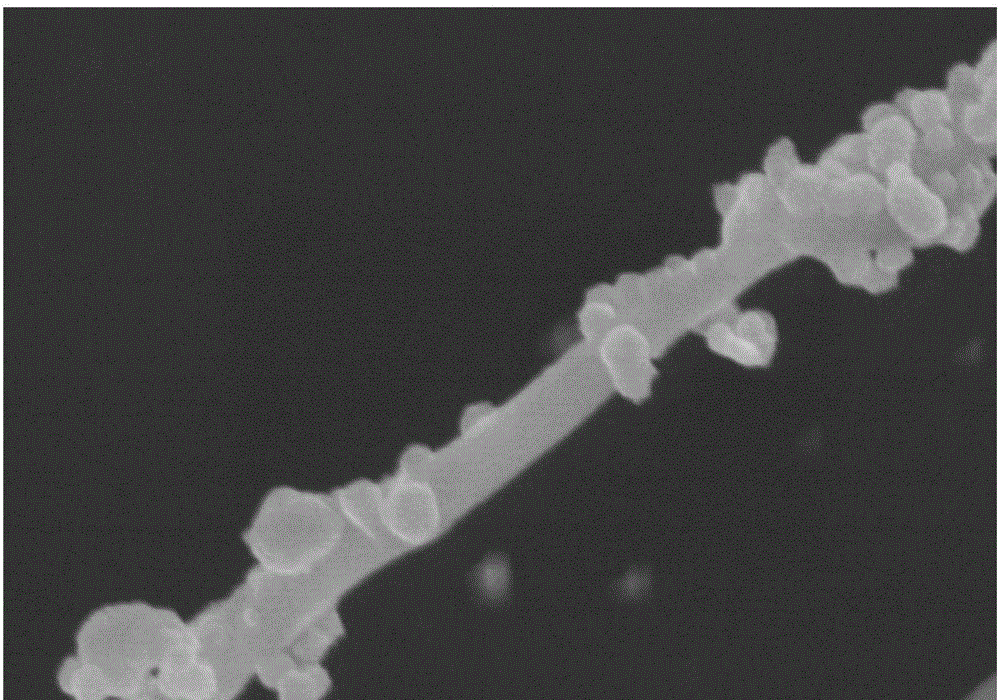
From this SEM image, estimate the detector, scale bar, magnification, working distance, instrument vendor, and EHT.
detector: SE(M)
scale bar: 1000 nm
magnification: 50 K X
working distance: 7.9 mm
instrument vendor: Hitachi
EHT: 5 kV